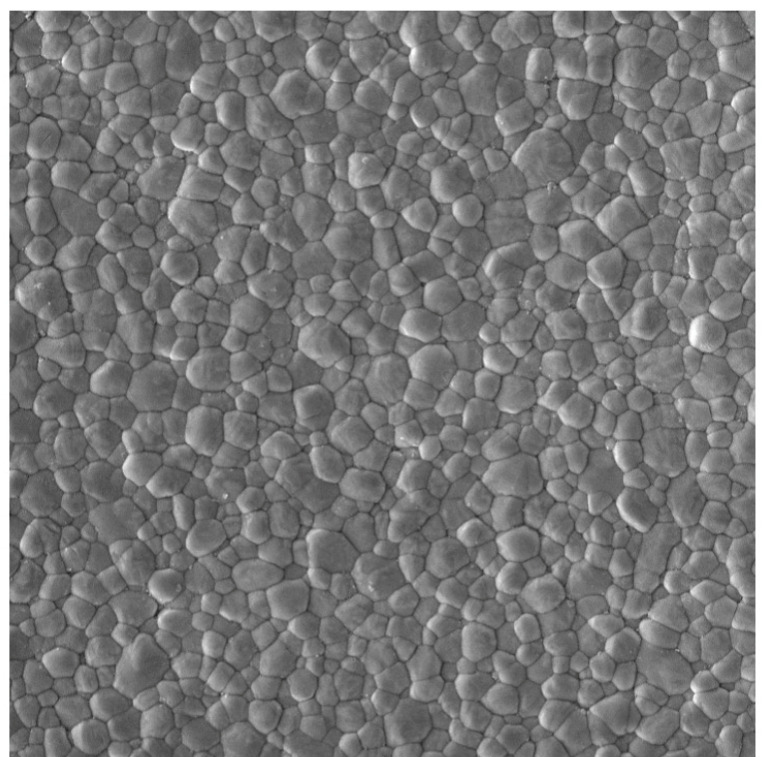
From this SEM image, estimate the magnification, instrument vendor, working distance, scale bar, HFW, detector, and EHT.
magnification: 20 K X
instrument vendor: Tescan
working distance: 5.34 mm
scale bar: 2000 nm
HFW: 13.8 µm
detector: In-Beam SE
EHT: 5 kV